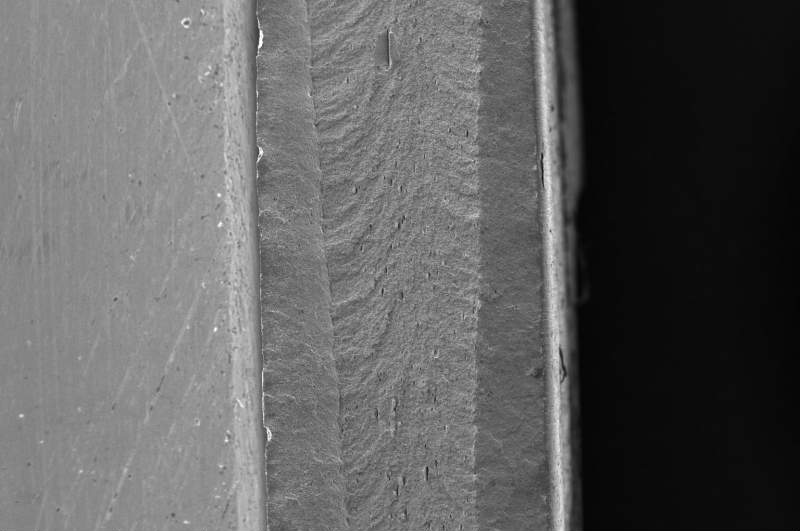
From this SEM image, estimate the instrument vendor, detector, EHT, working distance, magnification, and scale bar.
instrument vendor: FEI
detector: ETD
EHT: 20 kV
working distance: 10.7 mm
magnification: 0.1 K X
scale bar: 1000000 nm